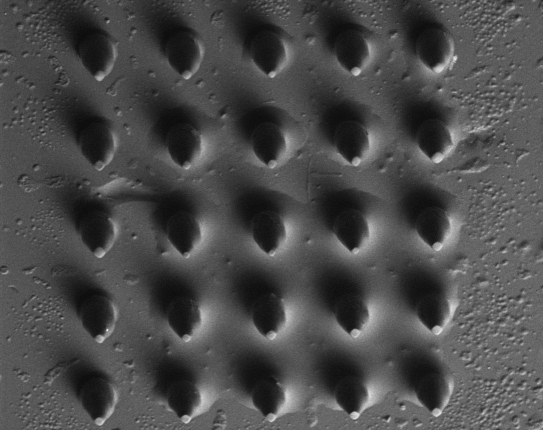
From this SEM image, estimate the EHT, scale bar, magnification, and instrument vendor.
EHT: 5 kV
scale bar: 500000 nm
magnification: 0.045 K X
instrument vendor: other